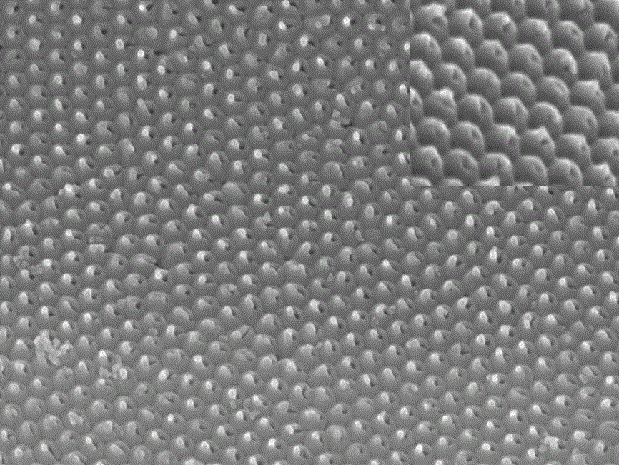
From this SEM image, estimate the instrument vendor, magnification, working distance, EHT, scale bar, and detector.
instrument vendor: JEOL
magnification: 50 K X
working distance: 13 mm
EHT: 15 kV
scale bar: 100 nm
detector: SEI